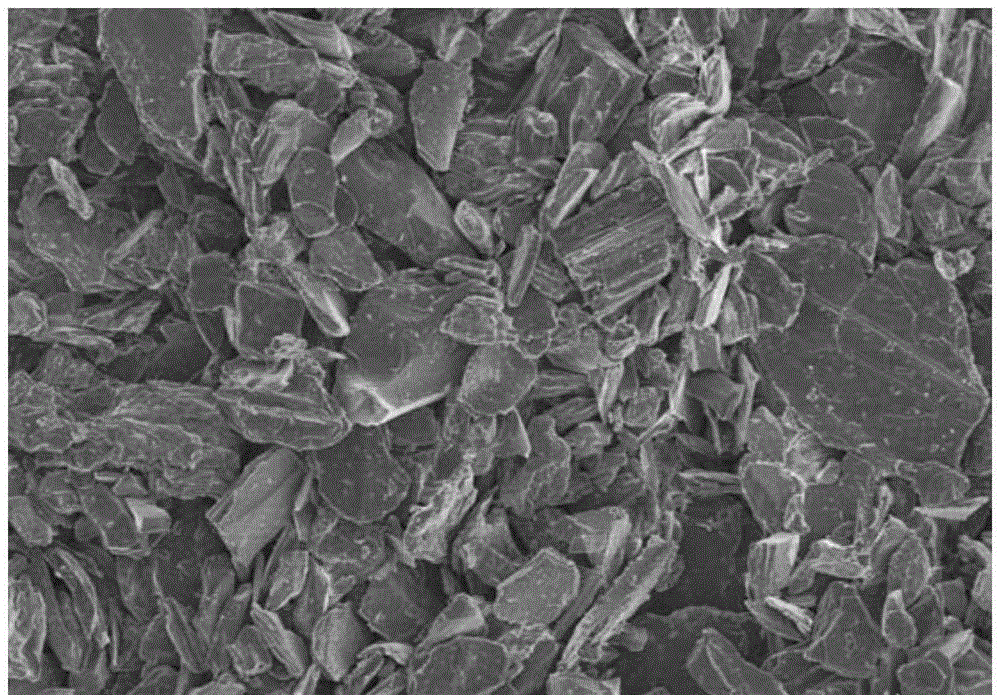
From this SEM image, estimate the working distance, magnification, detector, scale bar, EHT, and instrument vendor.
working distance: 8 mm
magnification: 1 K X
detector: SE(M)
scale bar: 50000 nm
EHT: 3 kV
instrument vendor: Hitachi